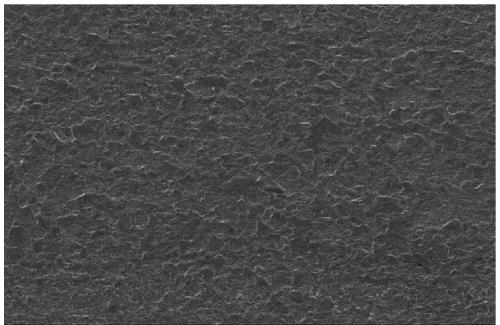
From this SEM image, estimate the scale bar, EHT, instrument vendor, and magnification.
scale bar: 50000 nm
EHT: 15 kV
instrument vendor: JEOL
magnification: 0.5 K X